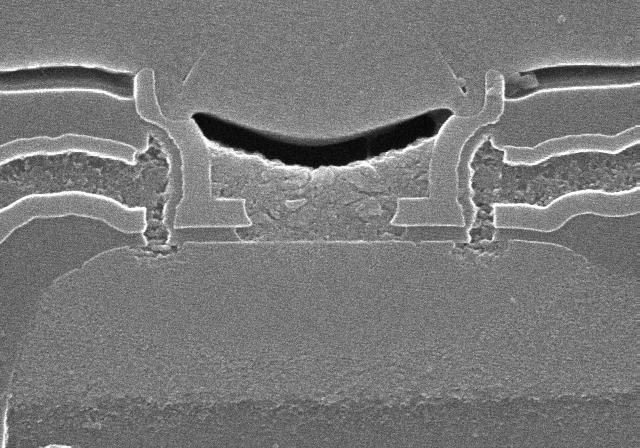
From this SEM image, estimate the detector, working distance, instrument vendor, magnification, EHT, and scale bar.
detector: SE(U)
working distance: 6.3 mm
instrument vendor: Hitachi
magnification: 50 K X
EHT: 10 kV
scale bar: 1000 nm